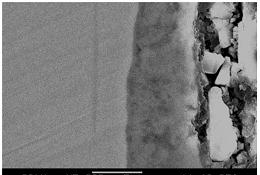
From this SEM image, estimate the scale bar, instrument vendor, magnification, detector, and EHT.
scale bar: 5000 nm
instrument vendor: JEOL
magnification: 5 K X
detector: SEI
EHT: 20 kV